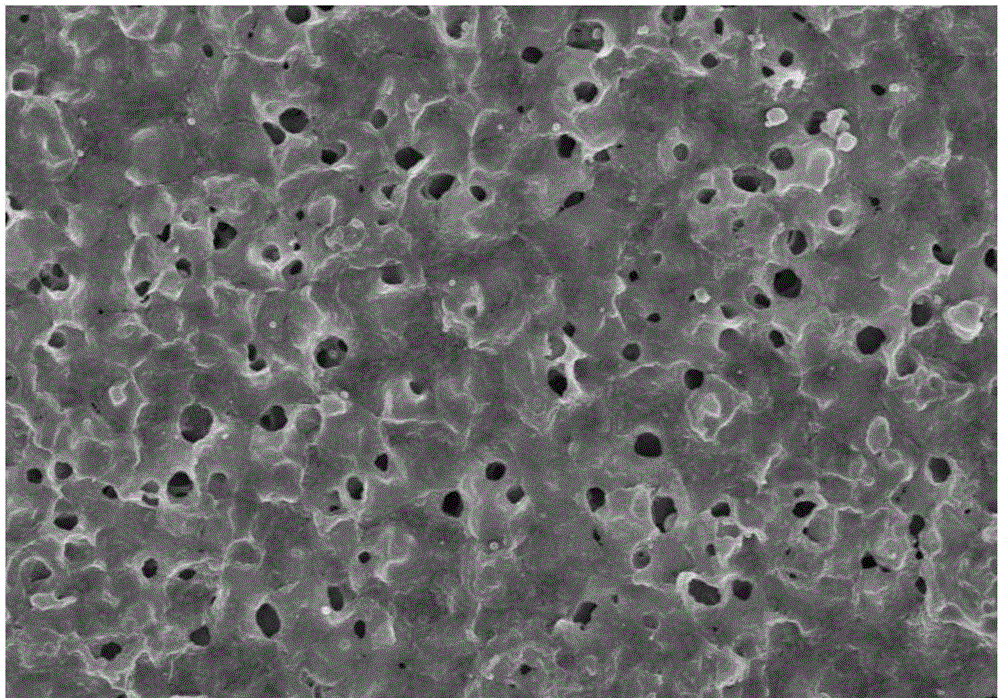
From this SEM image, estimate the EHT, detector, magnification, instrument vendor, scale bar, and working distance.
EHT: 15 kV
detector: SE(M)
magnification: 2 K X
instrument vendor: Hitachi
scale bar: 20000 nm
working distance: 6.4 mm